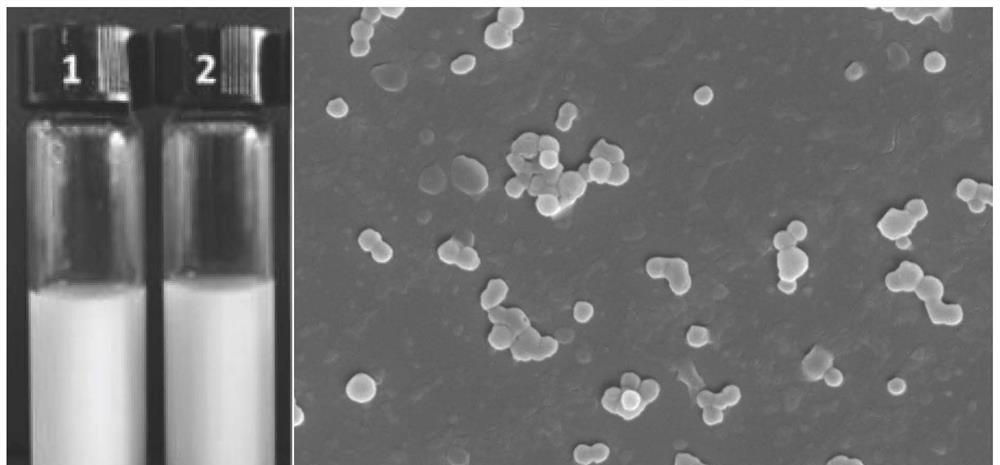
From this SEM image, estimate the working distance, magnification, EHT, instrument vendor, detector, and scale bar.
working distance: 6.4 mm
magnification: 30 K X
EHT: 5 kV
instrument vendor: Zeiss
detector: InLens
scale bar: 200 nm